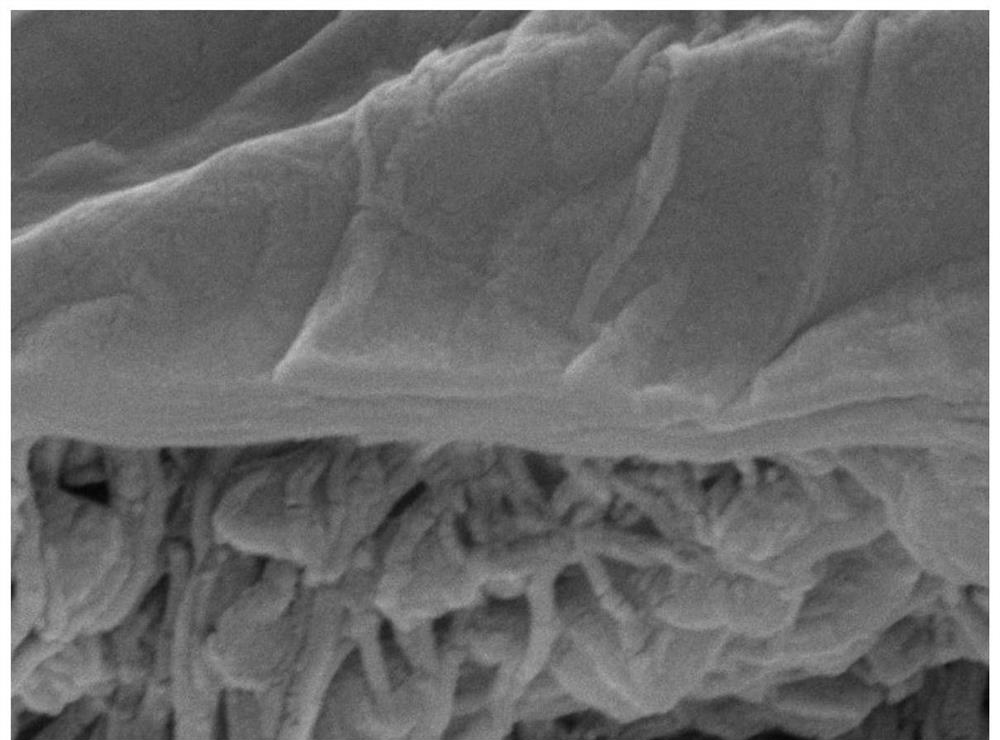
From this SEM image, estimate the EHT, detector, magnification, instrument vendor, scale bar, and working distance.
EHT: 15 kV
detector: LED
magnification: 40 K X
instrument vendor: JEOL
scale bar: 100 nm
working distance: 9.7 mm